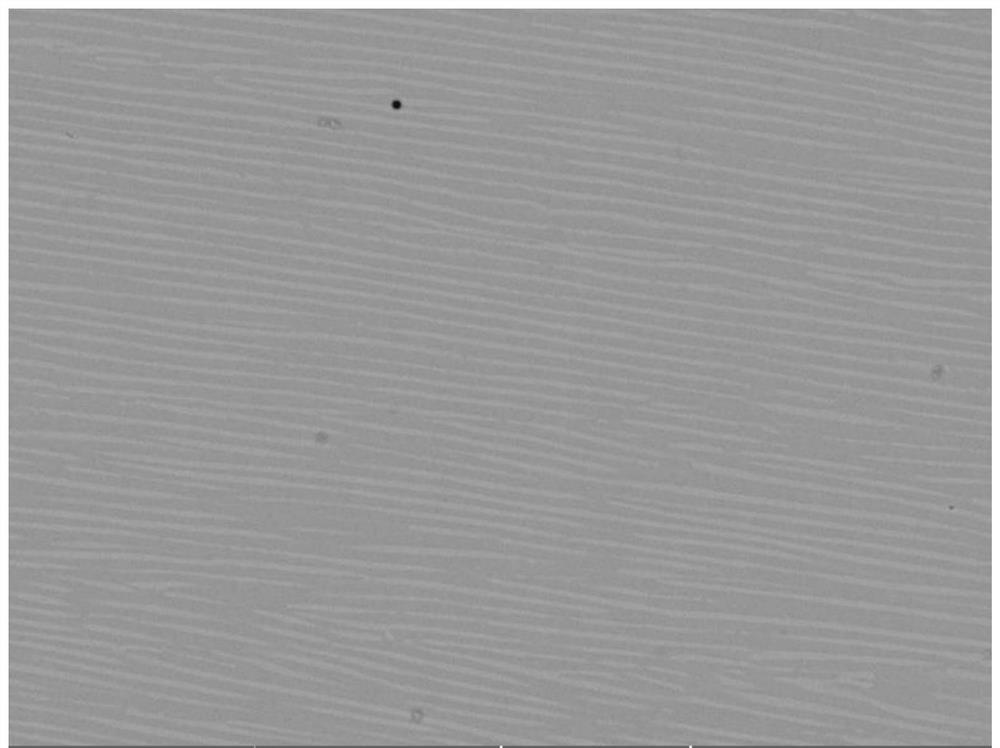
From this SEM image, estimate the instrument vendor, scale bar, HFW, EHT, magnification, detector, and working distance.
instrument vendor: Tescan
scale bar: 20000 nm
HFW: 104 µm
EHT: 20 kV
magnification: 2 K X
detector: BSE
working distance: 13.5 mm